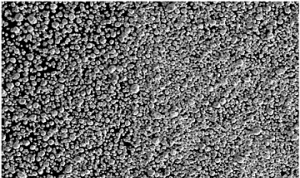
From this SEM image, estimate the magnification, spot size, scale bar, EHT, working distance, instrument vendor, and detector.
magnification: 0.3 K X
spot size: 3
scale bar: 500000 nm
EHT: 10 kV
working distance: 9.4 mm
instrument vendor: FEI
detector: ETD